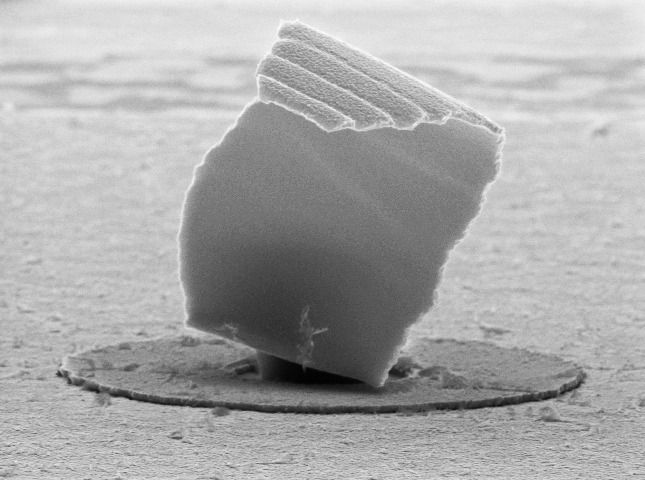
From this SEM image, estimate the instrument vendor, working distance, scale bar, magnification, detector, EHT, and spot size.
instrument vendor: FEI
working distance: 15.6 mm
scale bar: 1000 nm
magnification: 16.655 K X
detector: SE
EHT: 10 kV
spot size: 3